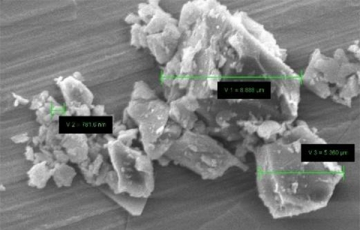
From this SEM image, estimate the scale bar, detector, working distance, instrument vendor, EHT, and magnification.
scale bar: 2000 nm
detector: SE1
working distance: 9.5 mm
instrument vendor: Zeiss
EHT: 10 kV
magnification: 5 K X